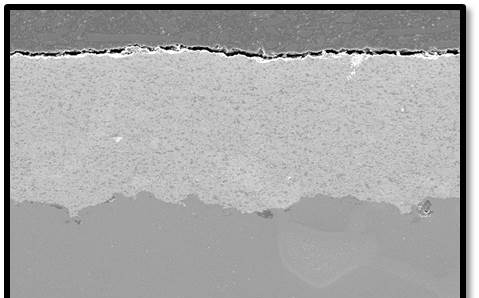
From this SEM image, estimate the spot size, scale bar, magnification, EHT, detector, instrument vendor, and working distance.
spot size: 3.6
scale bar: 200000 nm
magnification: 0.1 K X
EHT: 20 kV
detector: SE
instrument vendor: FEI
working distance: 10.5 mm